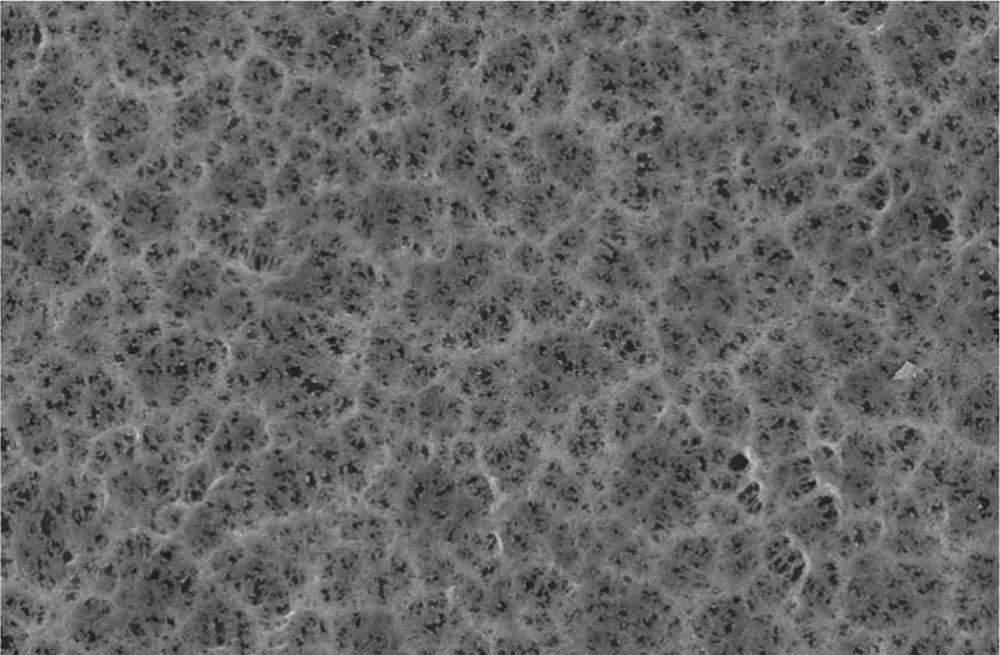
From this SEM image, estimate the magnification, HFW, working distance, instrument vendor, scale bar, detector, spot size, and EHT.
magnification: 10 K X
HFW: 41.4 µm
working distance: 7.3326 mm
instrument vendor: FEI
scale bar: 5000 nm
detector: ETD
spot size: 2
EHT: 10 kV